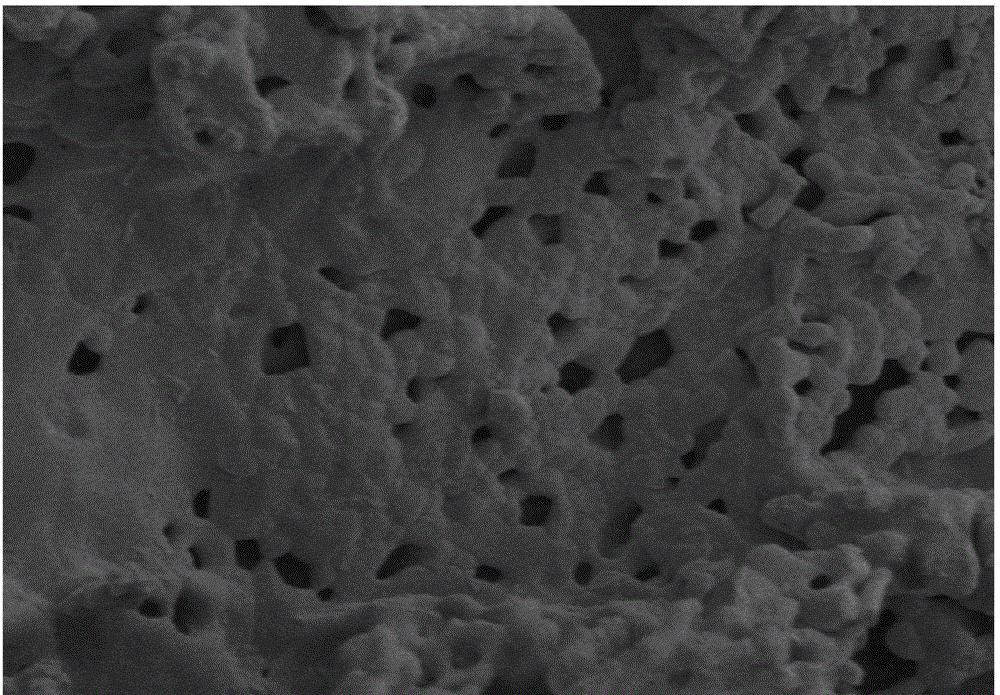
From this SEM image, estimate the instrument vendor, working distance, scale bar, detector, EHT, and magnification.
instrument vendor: Hitachi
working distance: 8.7 mm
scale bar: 3000 nm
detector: SE(M)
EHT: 3 kV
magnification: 15 K X